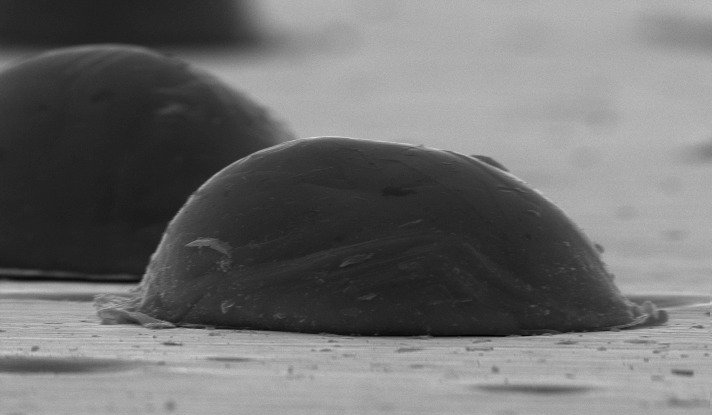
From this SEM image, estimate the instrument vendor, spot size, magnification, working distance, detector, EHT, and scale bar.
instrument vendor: FEI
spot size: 4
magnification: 8 K X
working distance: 9.5 mm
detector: SE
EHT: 20 kV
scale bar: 10000 nm